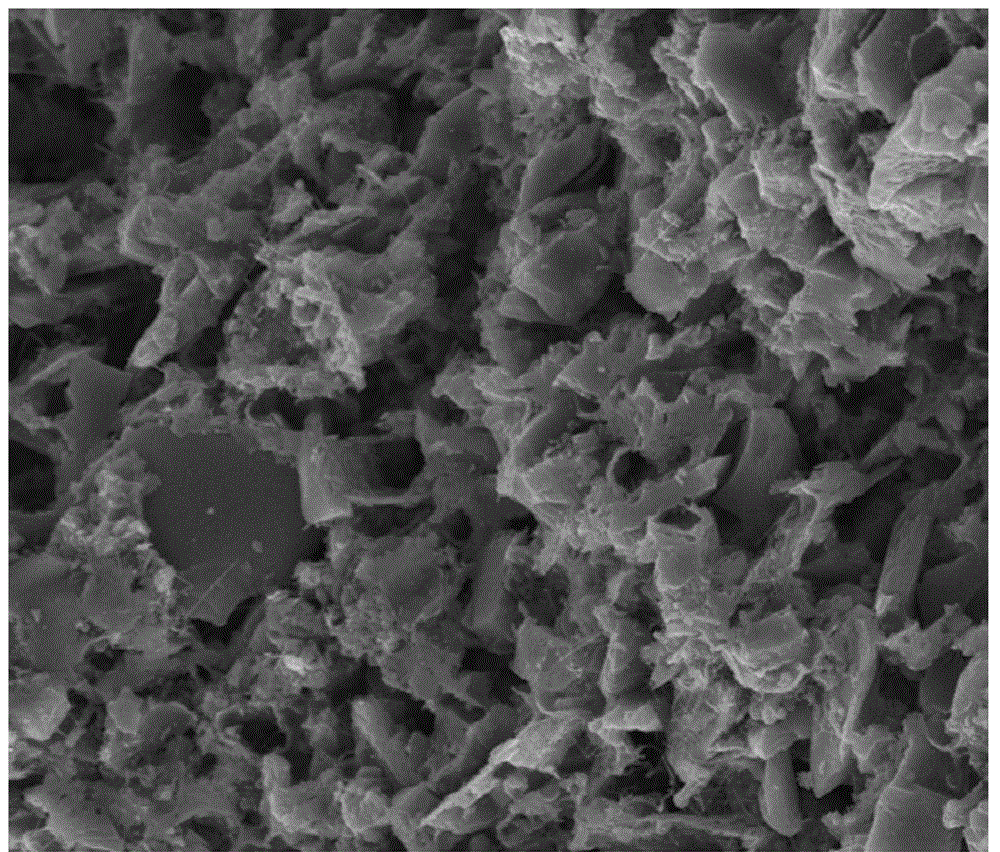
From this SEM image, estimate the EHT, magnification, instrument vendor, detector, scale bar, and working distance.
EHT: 20 kV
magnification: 5 K X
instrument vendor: FEI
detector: ETD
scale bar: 500000 nm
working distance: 14.9 mm